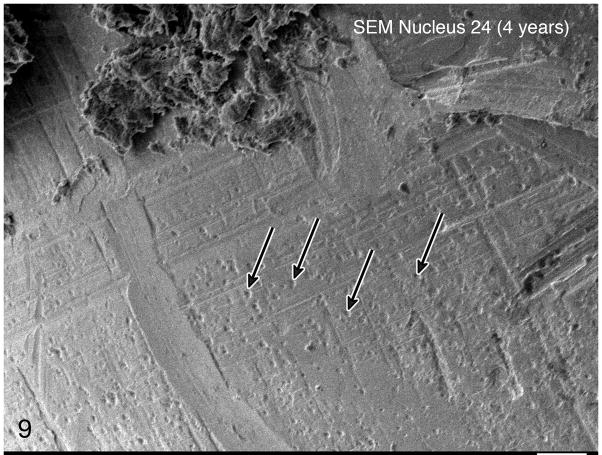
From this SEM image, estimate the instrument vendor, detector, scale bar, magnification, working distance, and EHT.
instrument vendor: JEOL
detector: LEI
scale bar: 10000 nm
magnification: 1 K X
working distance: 7.7 mm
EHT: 5 kV